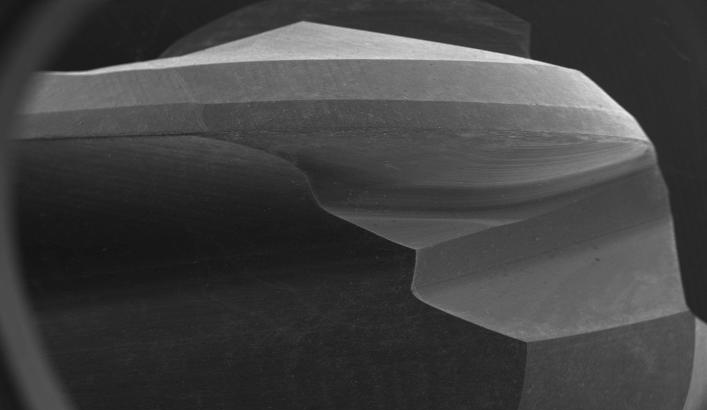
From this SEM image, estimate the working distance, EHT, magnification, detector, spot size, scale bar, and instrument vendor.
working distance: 25.4 mm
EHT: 20 kV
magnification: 0.008 K X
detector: SE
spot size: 5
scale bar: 2000000 nm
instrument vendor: FEI